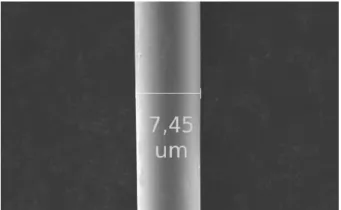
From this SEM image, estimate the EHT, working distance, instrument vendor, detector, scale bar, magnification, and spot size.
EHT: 20 kV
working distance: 10 mm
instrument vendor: FEI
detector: SE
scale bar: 10000 nm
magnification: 3.2 K X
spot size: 2